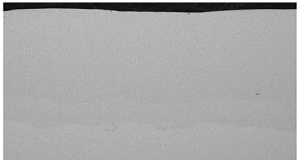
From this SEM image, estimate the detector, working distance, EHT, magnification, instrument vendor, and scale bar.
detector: BSE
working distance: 15.74 mm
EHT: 15 kV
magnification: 0.07 K X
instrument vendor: Tescan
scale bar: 1000000 nm